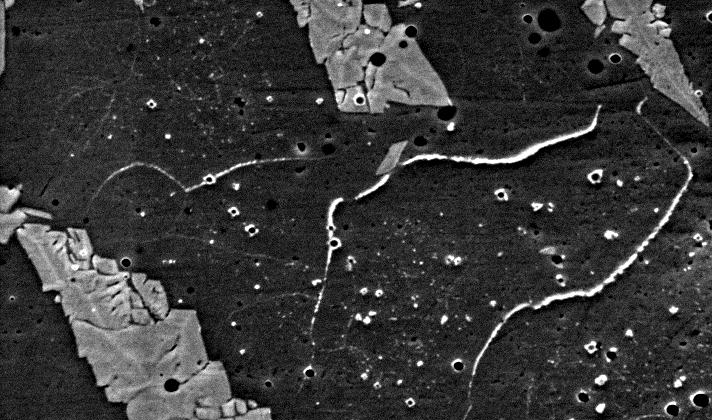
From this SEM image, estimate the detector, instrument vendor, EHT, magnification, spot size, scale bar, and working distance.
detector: SE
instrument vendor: FEI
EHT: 20 kV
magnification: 4 K X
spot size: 5.4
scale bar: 10000 nm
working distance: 10.1 mm